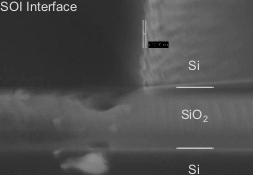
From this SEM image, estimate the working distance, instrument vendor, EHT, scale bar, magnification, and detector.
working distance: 3.7 mm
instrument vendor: Zeiss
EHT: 5 kV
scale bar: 200 nm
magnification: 148.79 K X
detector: InLens